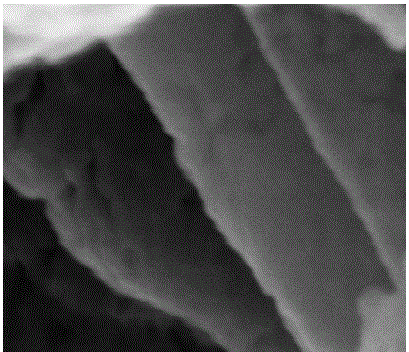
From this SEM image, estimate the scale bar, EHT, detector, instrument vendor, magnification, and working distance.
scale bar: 100 nm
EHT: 15 kV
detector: SE(U)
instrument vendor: Hitachi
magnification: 350 K X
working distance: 10.1 mm